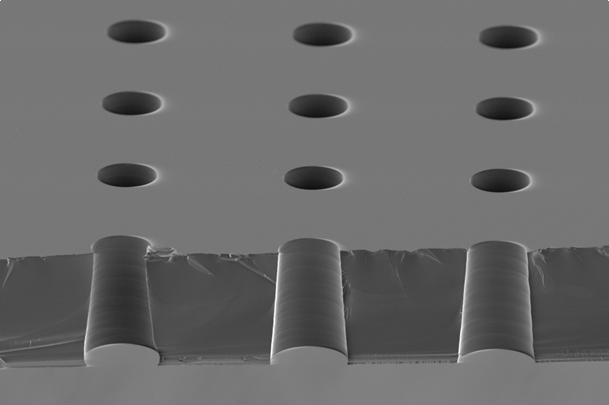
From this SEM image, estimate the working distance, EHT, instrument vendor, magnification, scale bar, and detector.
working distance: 33 mm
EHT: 8 kV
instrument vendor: JEOL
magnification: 0.4 K X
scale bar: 50000 nm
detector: SEI